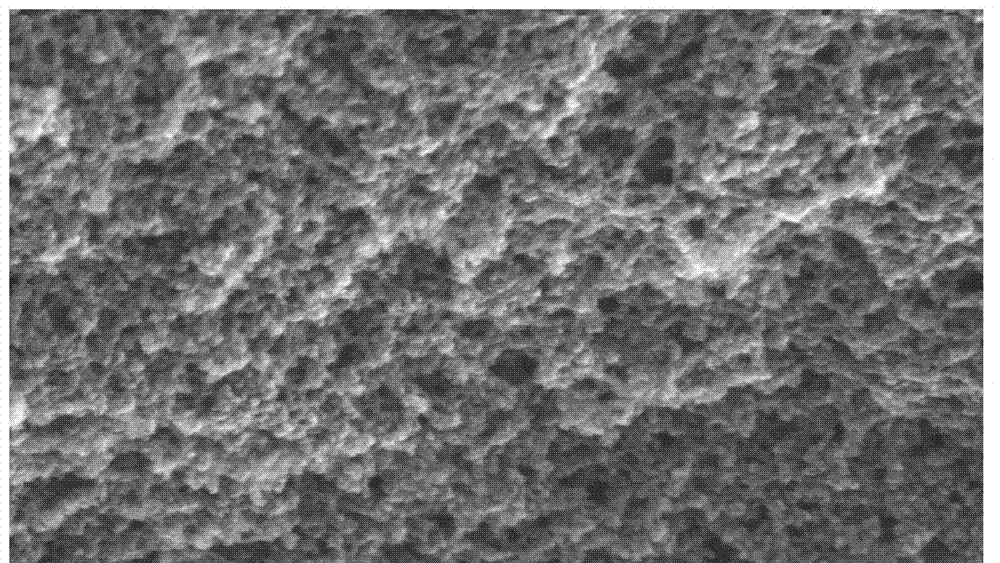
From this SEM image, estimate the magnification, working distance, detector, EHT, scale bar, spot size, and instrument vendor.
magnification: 80 K X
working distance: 5 mm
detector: TLD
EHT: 20 kV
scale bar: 200 nm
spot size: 4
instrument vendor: FEI